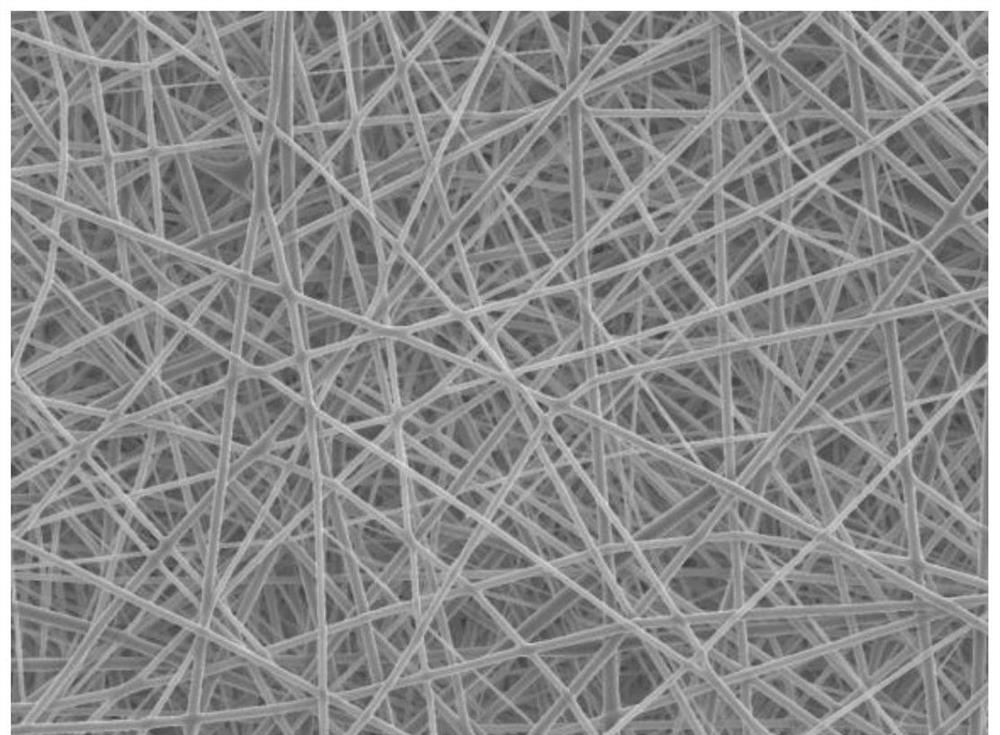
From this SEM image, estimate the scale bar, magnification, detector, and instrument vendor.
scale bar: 10000 nm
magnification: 2 K X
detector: LED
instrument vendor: JEOL